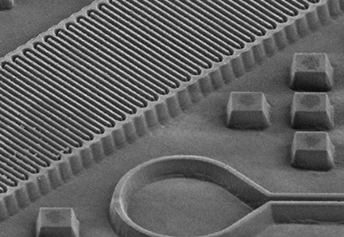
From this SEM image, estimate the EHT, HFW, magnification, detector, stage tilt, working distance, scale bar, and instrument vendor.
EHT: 5 kV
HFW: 280 µm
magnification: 0.533 K X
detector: ETD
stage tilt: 52°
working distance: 9.9 mm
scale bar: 100000 nm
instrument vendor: FEI